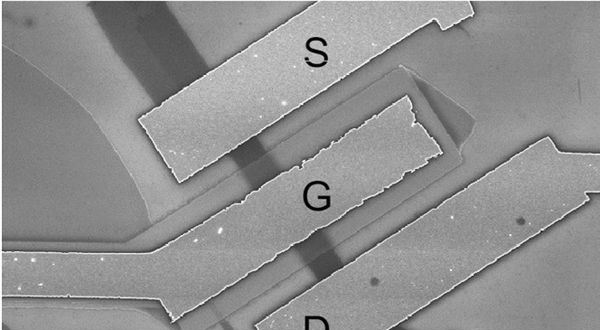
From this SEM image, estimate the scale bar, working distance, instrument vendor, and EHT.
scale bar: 3000 nm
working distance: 5 mm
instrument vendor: Zeiss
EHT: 5 kV